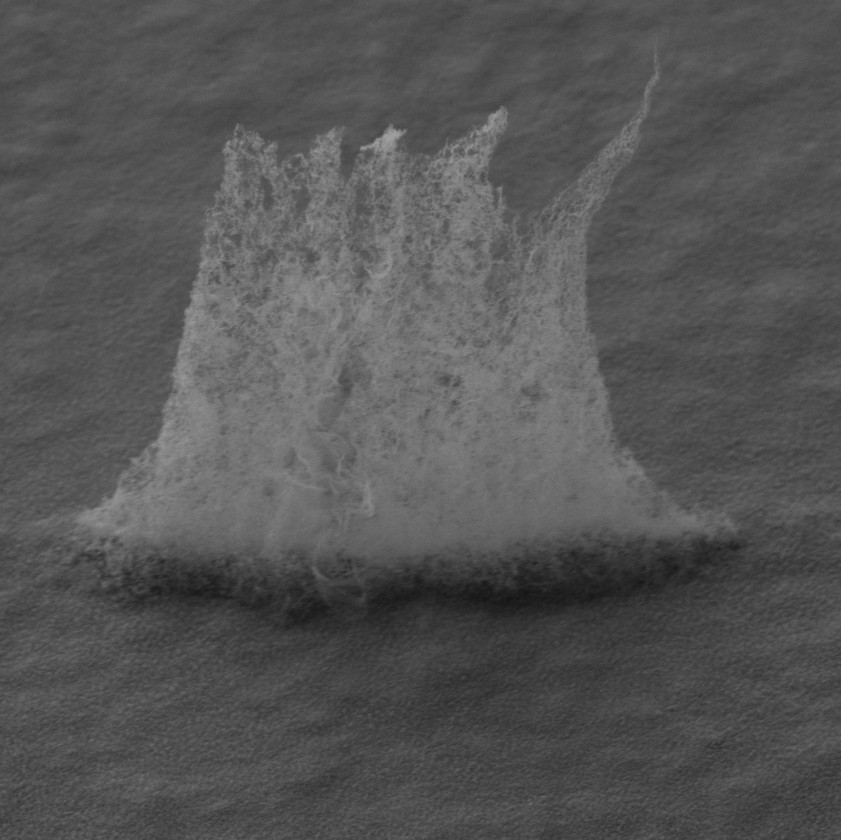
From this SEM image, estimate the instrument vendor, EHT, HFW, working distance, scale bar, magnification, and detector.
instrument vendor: Tescan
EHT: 30 kV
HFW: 82.9 µm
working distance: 14 mm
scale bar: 20000 nm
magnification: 5.02 K X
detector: SE